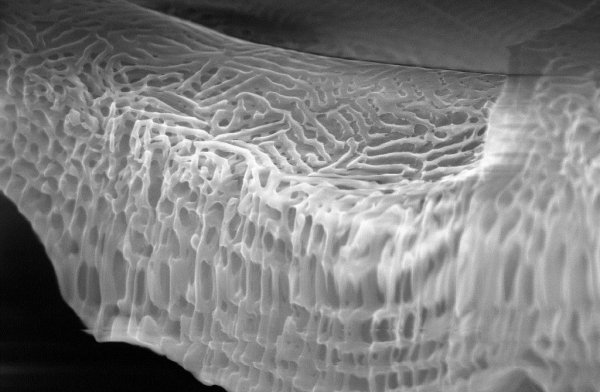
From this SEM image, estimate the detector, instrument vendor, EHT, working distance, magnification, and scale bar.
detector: InLens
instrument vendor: Zeiss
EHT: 10 kV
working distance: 3 mm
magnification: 58.48 K X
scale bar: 1000 nm